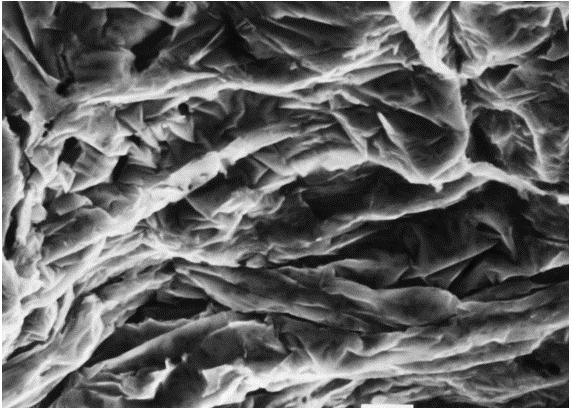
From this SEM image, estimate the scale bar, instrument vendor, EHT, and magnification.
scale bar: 10000 nm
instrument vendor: JEOL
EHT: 25 kV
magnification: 1 K X